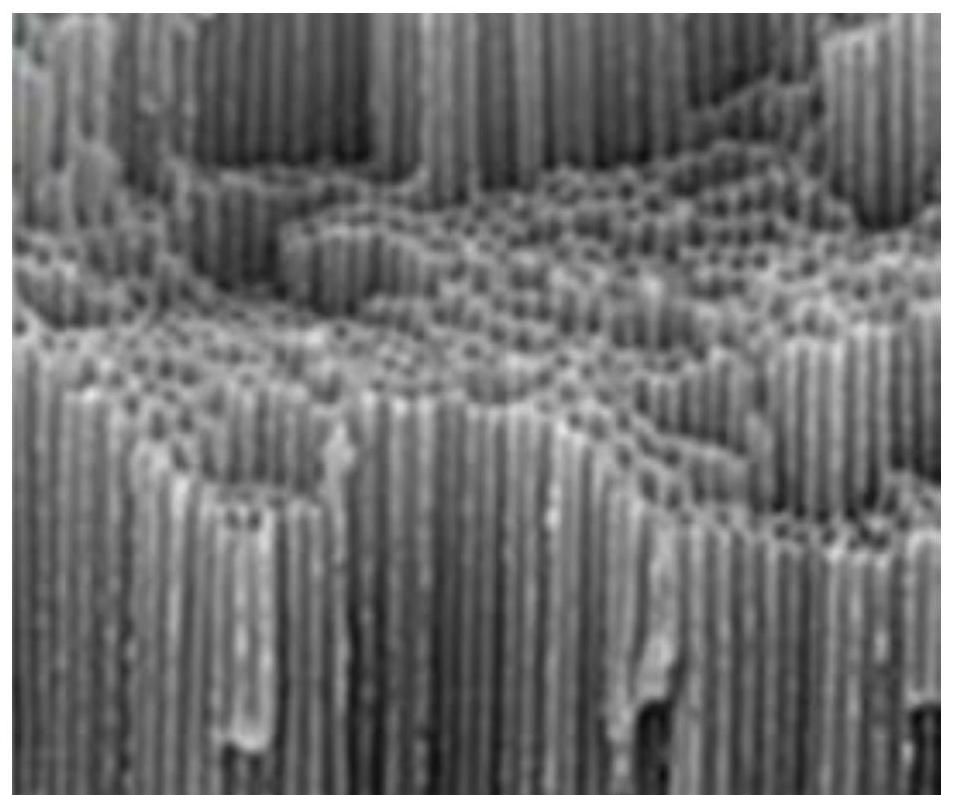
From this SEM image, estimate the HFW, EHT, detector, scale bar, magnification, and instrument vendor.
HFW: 54.2 µm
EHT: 5 kV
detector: BSD Full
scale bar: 10000 nm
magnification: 5 K X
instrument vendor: Thermo